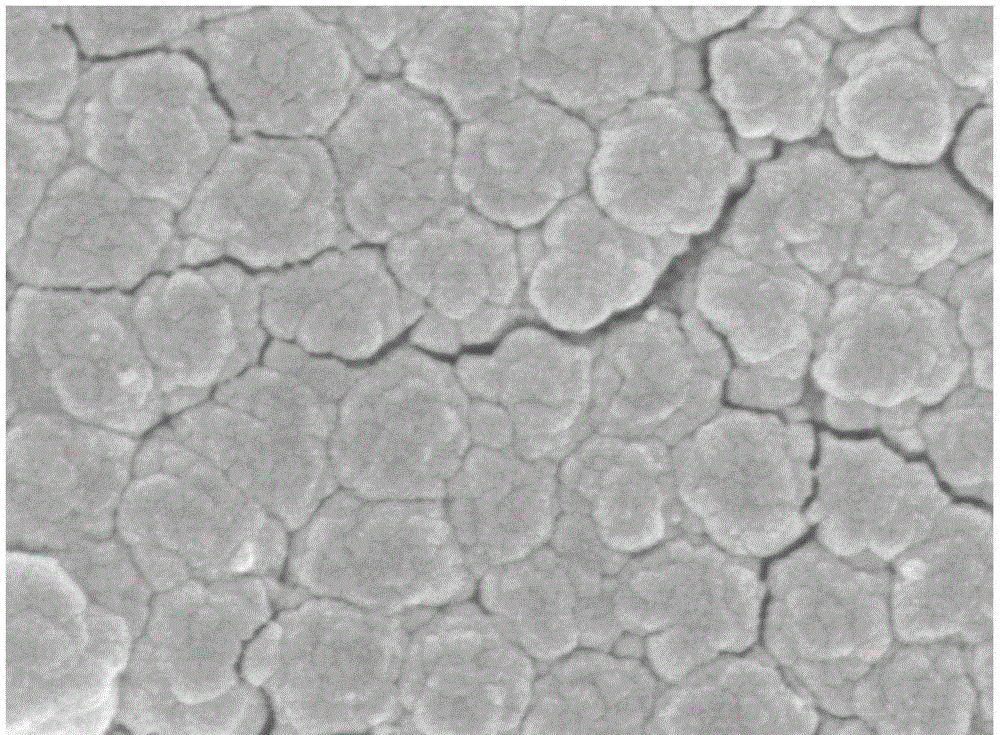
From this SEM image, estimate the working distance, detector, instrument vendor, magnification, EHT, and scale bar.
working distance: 8.4 mm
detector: SEI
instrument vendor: JEOL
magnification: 40 K X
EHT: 5 kV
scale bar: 100 nm